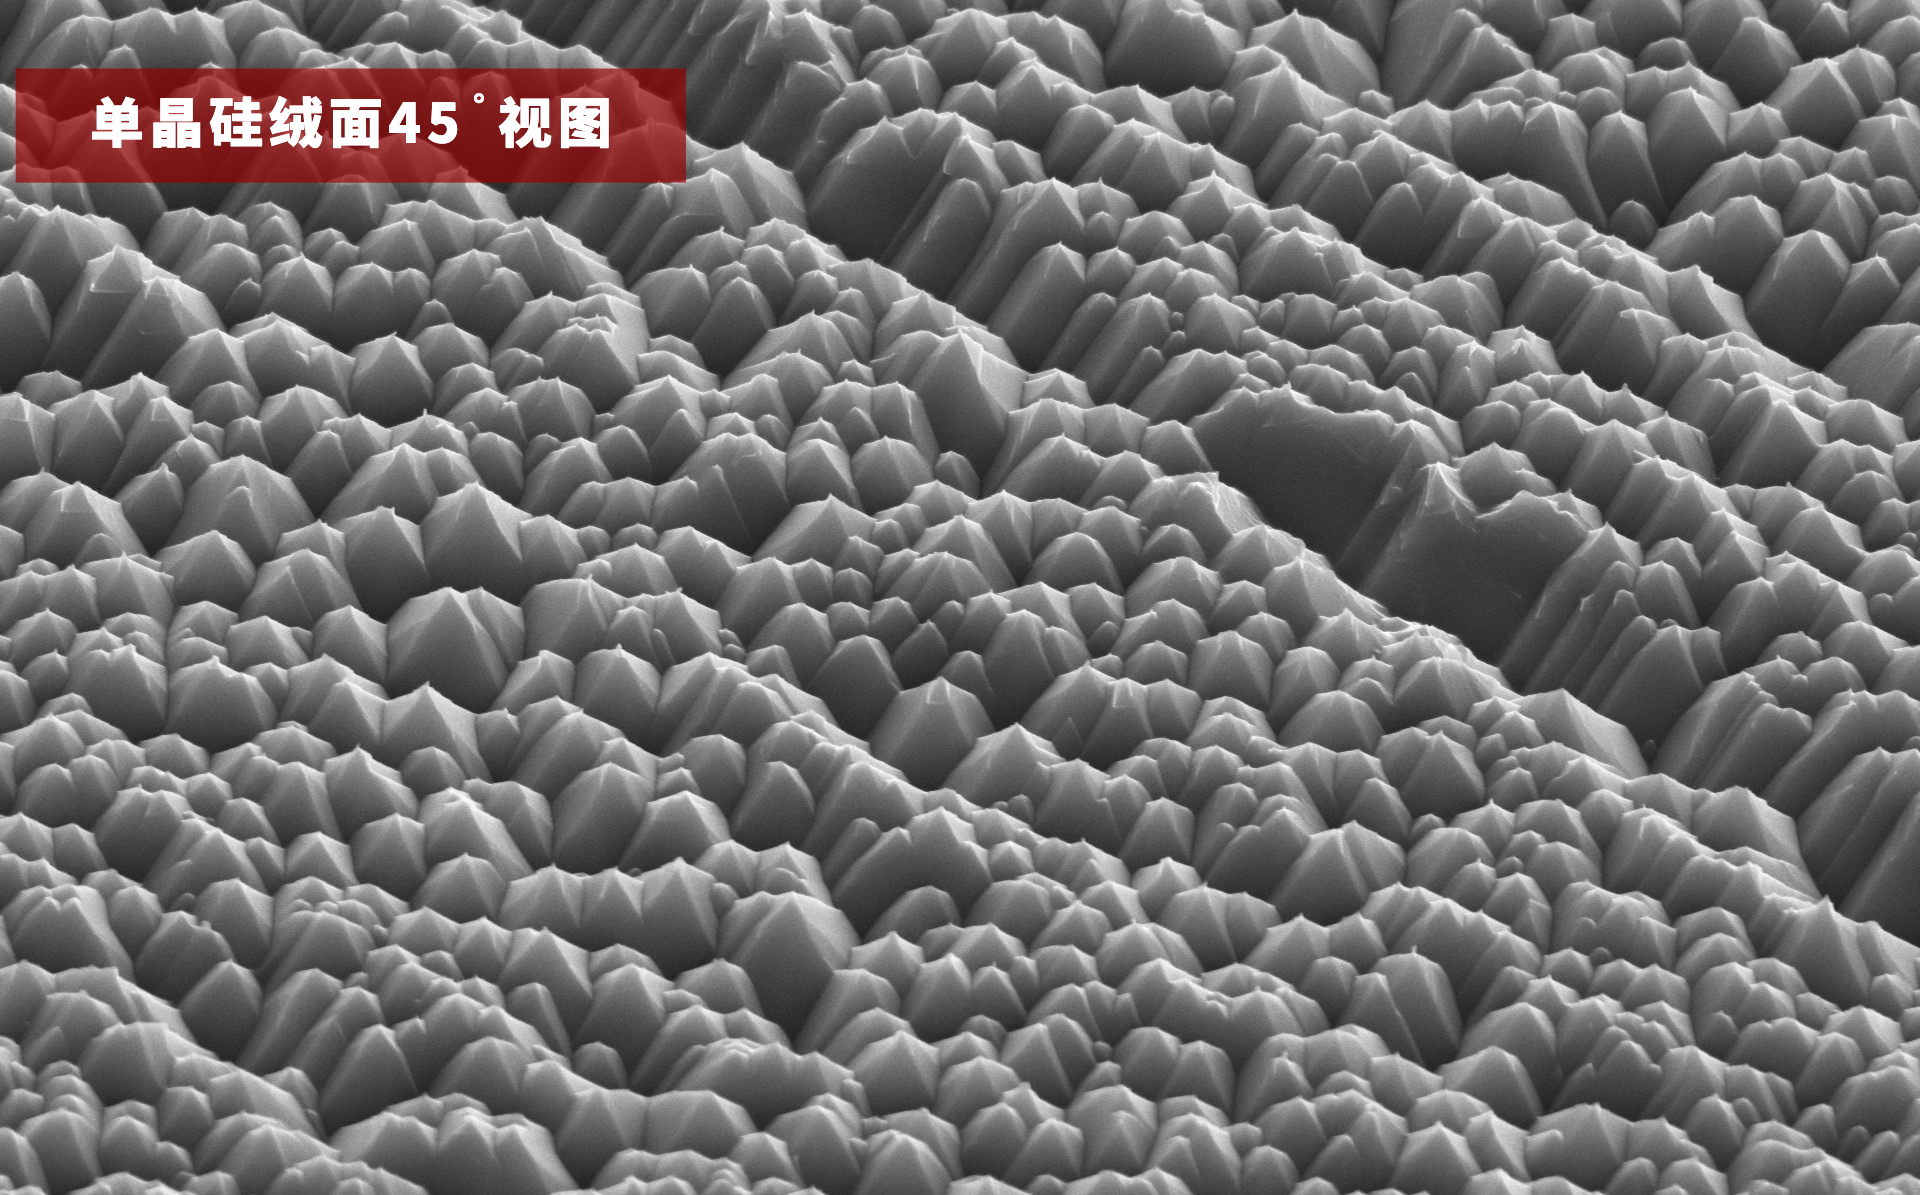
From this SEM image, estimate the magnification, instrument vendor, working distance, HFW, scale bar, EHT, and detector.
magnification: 5 K X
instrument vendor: other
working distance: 9.434 mm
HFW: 25.4 µm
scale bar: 8000 nm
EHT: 10 kV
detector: SED